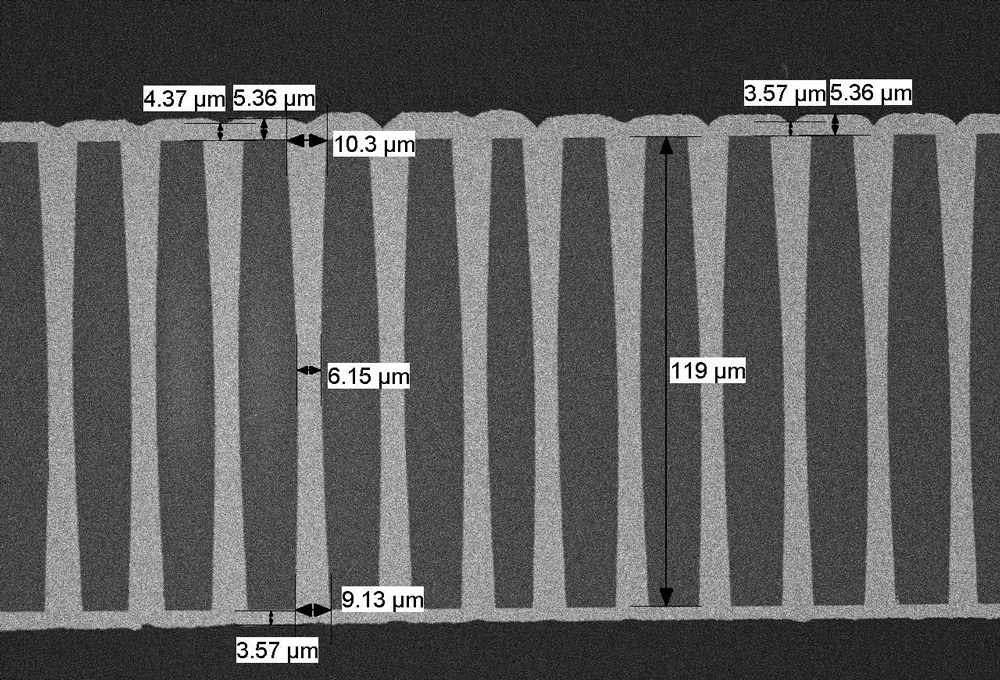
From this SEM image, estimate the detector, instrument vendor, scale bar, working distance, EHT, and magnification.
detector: HA3(T)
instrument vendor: Hitachi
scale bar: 100000 nm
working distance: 8.1 mm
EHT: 3 kV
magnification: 0.5 K X